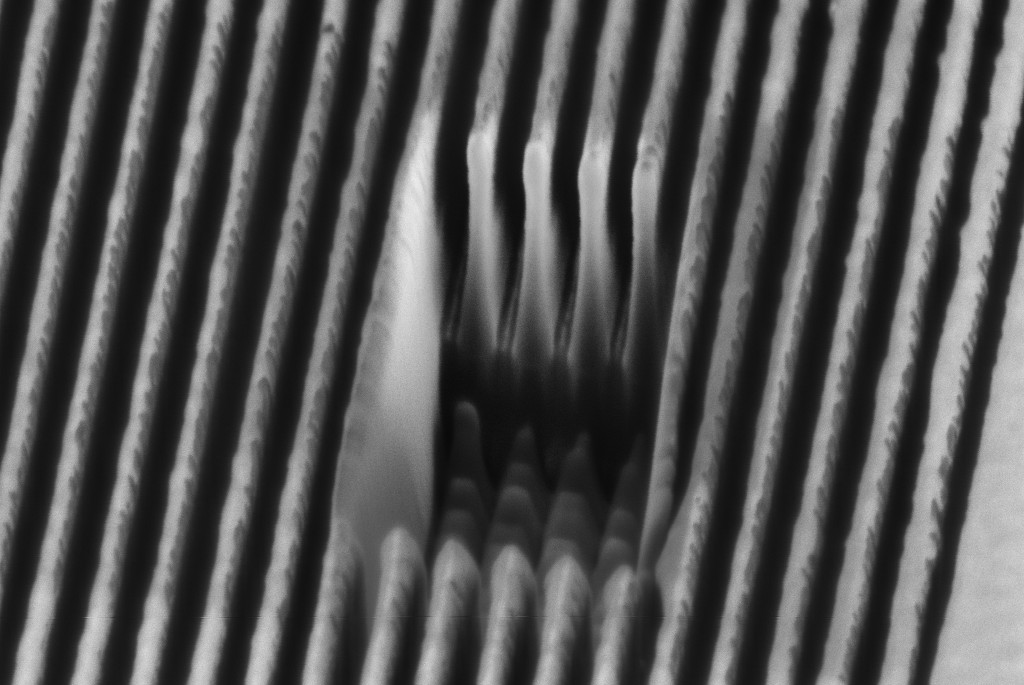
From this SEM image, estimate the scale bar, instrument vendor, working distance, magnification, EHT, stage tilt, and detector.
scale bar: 200 nm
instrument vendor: Zeiss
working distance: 5 mm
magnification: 21.56 K X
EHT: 2 kV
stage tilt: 54°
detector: InLens